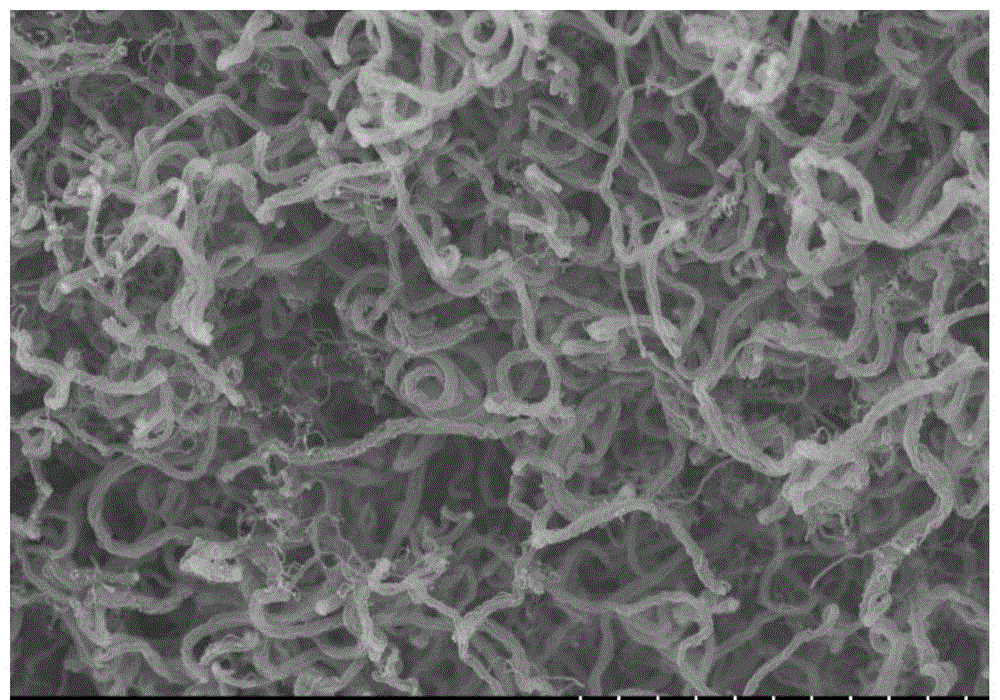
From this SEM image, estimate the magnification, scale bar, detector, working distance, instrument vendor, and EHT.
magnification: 10 K X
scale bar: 5000 nm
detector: SE(UL)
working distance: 12.1 mm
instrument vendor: Hitachi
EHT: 10 kV